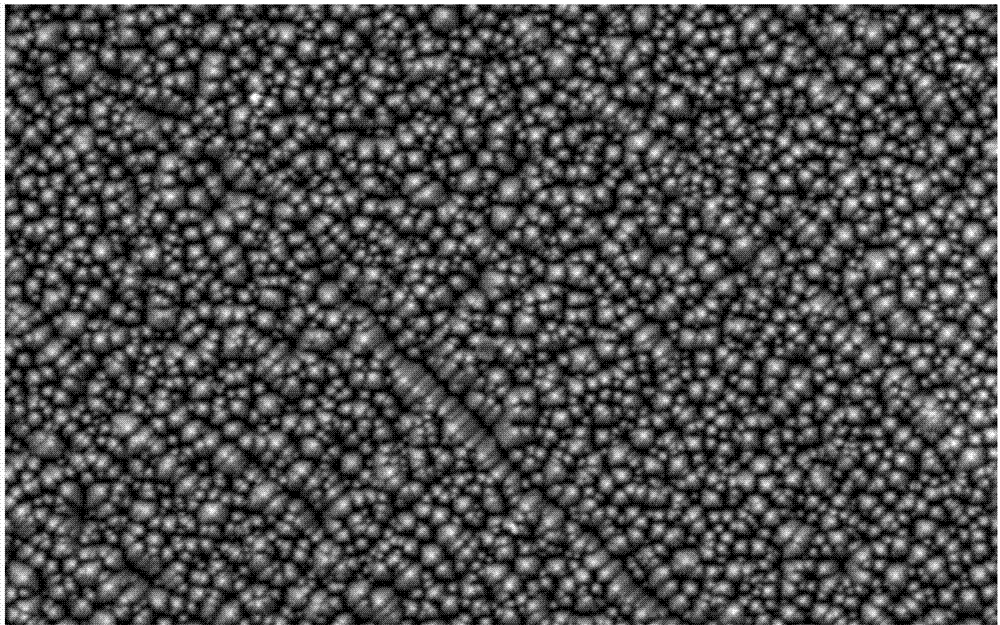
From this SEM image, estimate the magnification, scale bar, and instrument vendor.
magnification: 1.5 K X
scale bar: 10000 nm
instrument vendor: JEOL